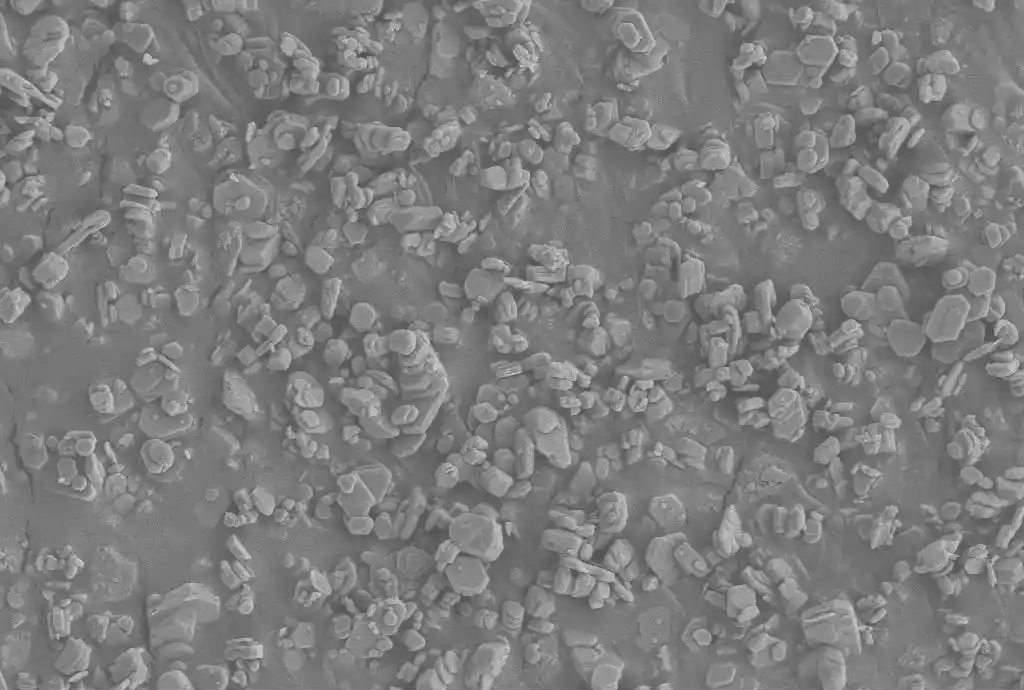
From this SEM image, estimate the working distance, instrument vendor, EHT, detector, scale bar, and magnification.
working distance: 8.9 mm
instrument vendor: Zeiss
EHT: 3 kV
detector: SE2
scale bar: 1000 nm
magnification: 5 K X